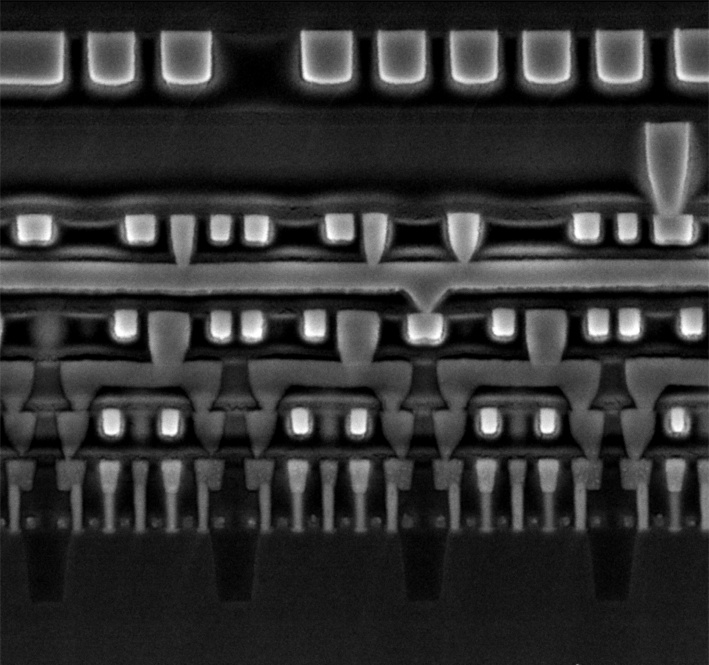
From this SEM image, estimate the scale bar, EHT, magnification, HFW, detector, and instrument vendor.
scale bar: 200 nm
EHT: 5 kV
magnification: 100 K X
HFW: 2.9 µm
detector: SE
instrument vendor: other